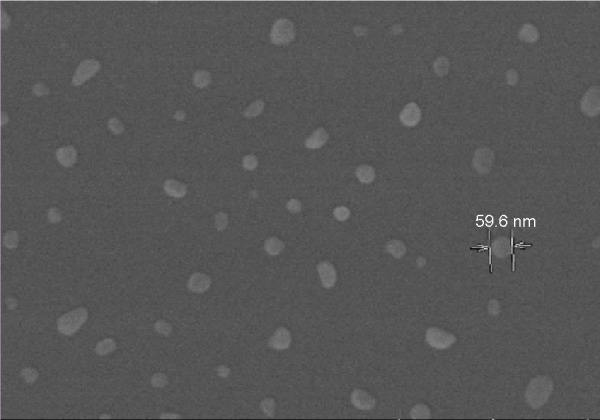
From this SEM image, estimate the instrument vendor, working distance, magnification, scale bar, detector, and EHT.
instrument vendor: Hitachi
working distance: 2.9 mm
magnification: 80 K X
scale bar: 500 nm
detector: SE(U)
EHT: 3 kV